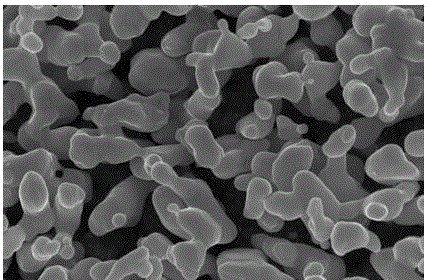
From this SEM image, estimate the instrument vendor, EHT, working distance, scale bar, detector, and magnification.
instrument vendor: FEI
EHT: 15 kV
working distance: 5 mm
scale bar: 2000 nm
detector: SE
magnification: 100 K X